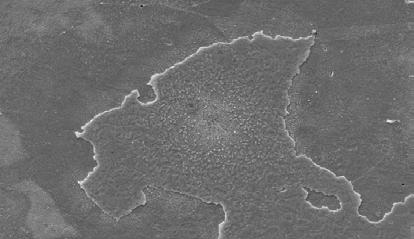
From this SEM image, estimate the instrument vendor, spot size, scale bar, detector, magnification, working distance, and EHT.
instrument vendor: FEI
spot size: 3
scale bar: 50000 nm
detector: SE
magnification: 1 K X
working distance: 10.2 mm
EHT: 20 kV